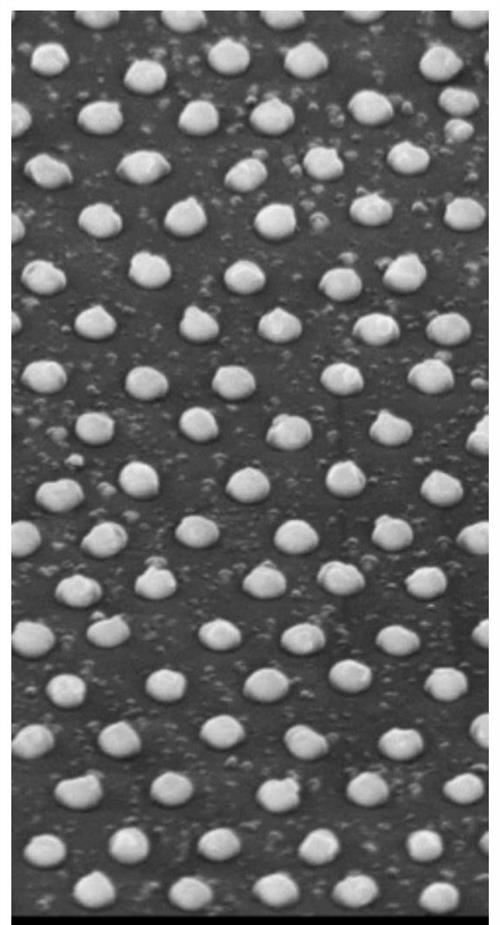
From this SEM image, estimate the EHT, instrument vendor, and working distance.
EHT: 10 kV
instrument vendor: Hitachi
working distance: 12.8 mm